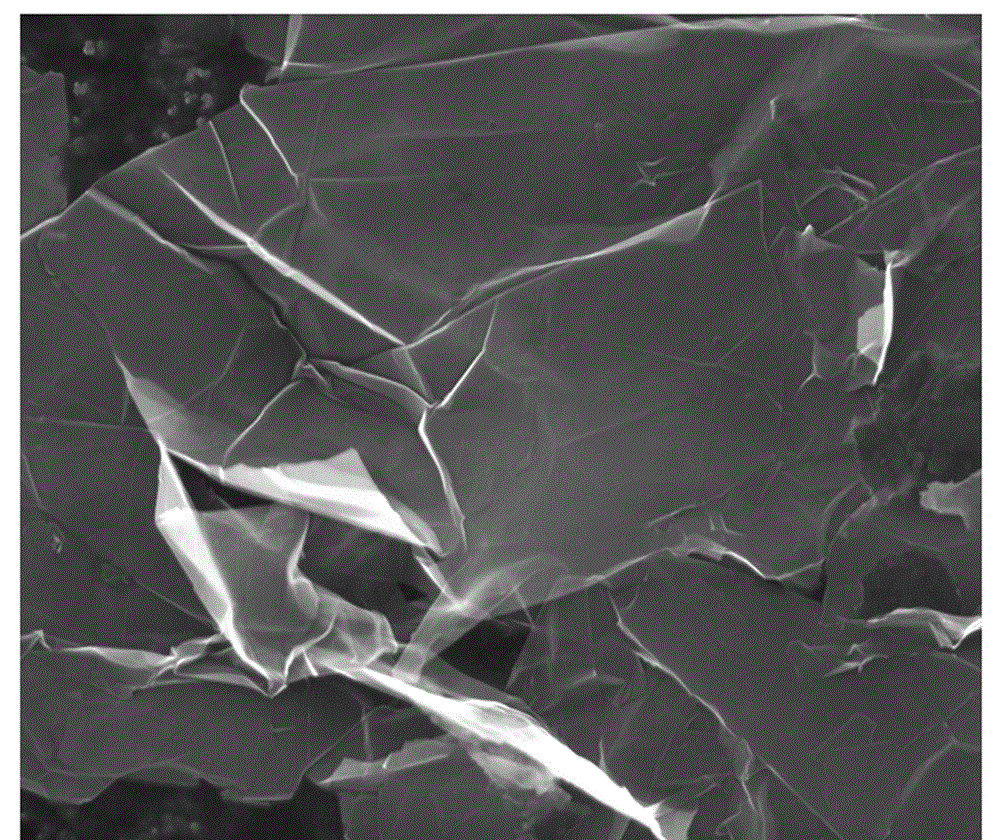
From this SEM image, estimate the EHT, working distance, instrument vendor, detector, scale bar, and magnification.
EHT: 20 kV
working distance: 8.3 mm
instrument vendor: FEI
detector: ETD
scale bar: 5000 nm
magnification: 25 K X